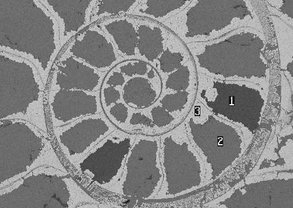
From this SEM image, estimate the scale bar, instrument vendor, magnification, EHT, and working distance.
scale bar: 500000 nm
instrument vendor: Zeiss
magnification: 0.045 K X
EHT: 20 kV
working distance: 26 mm